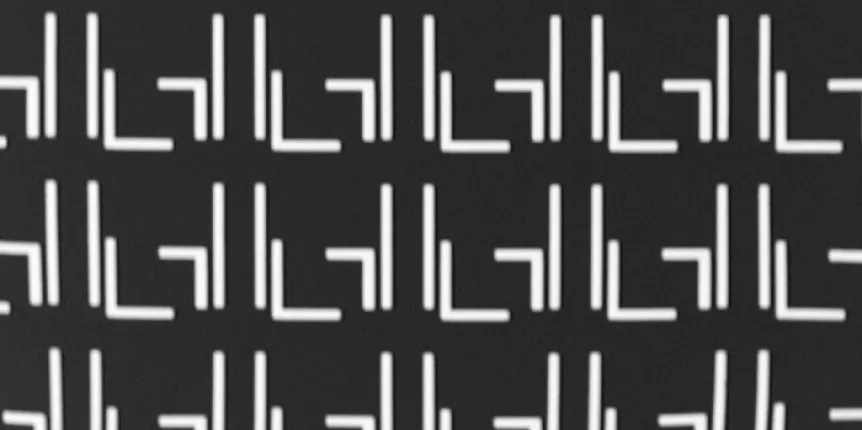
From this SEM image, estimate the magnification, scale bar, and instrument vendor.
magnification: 0.485 K X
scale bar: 240000 nm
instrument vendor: Thermo